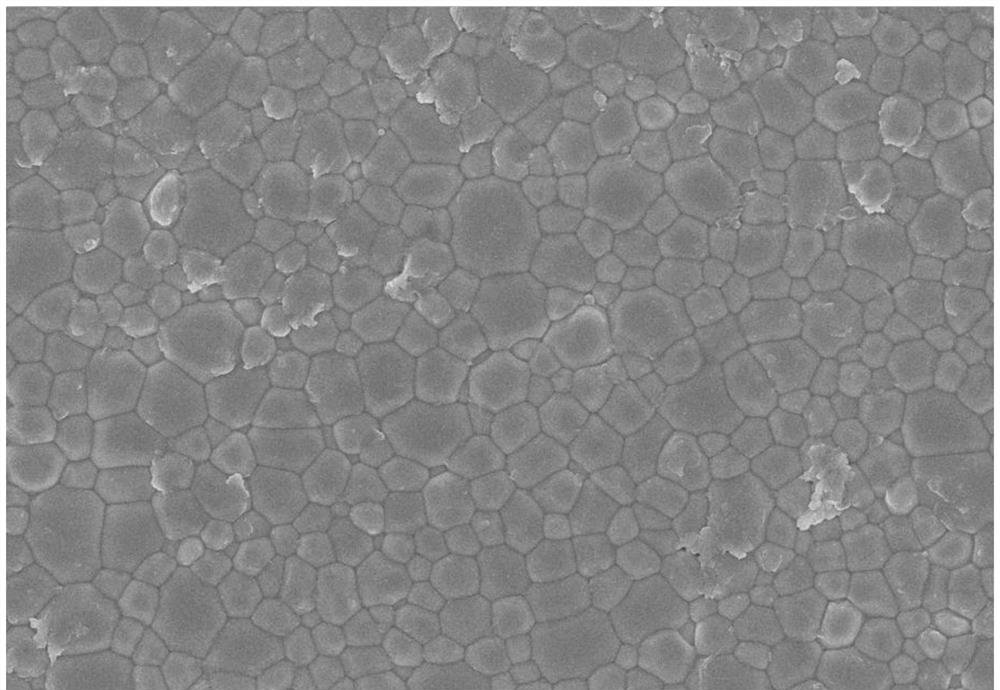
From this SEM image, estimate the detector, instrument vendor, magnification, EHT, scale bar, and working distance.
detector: InLens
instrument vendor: Zeiss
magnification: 10 K X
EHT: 5 kV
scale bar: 1000 nm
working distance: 6.6 mm